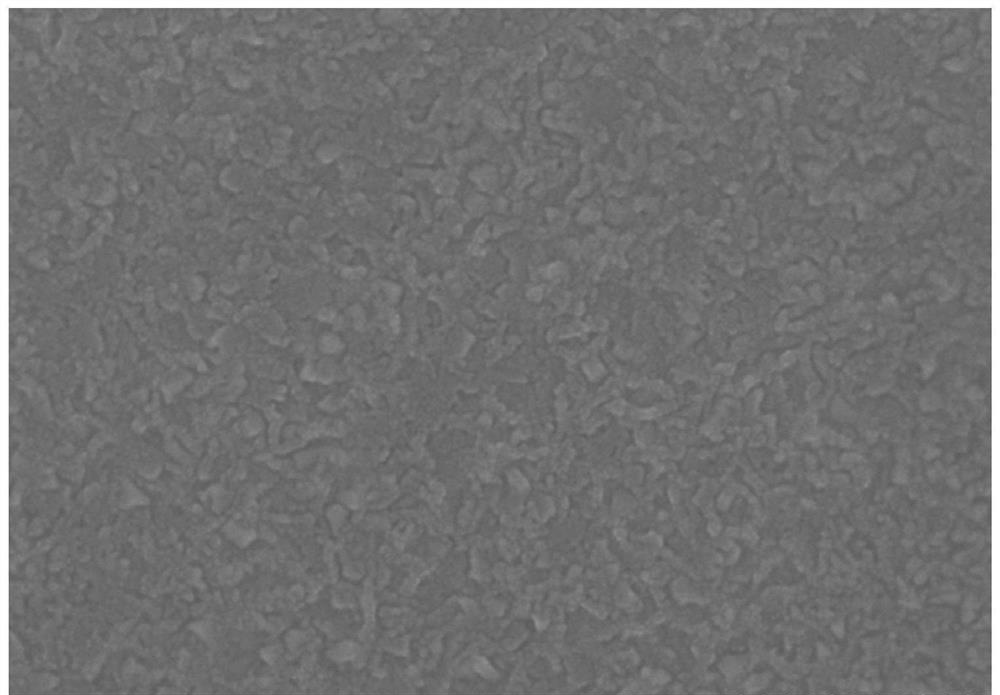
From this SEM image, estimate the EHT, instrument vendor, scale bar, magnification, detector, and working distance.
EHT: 10 kV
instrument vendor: Hitachi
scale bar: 400 nm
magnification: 200 K X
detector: SE(U)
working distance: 11.8 mm